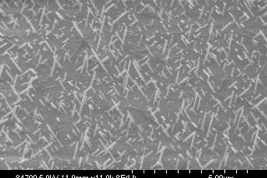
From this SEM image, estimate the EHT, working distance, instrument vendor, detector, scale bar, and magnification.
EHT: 5 kV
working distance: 11.9 mm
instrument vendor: Hitachi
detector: SE(U)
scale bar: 5000 nm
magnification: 11 K X